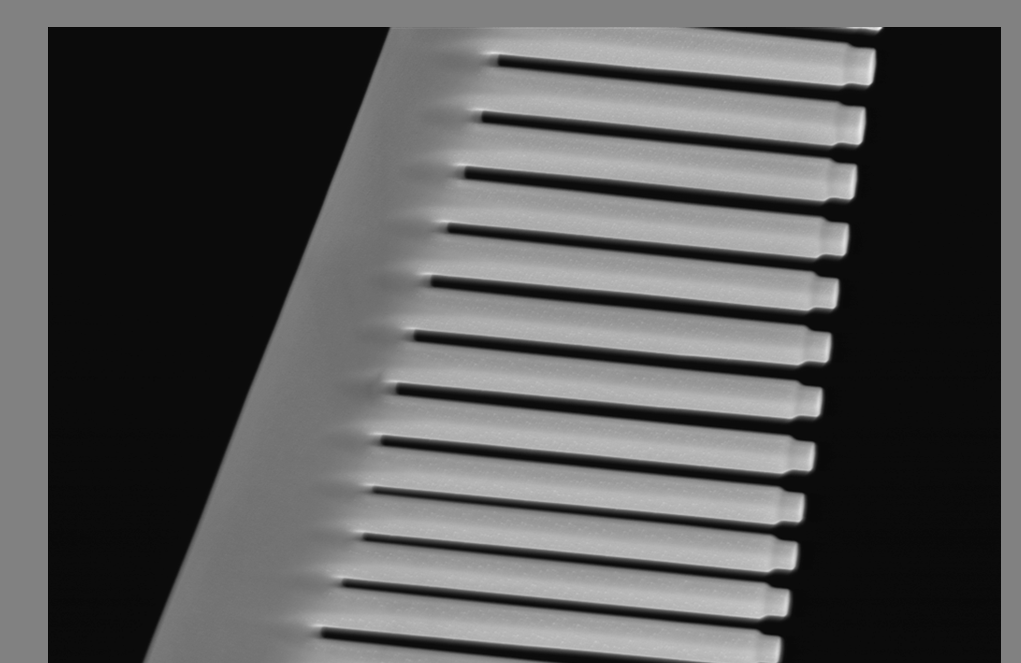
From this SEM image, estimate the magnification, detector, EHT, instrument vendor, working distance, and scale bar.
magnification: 10.67 K X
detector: InLens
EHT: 10 kV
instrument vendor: Zeiss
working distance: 4 mm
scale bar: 2000 nm